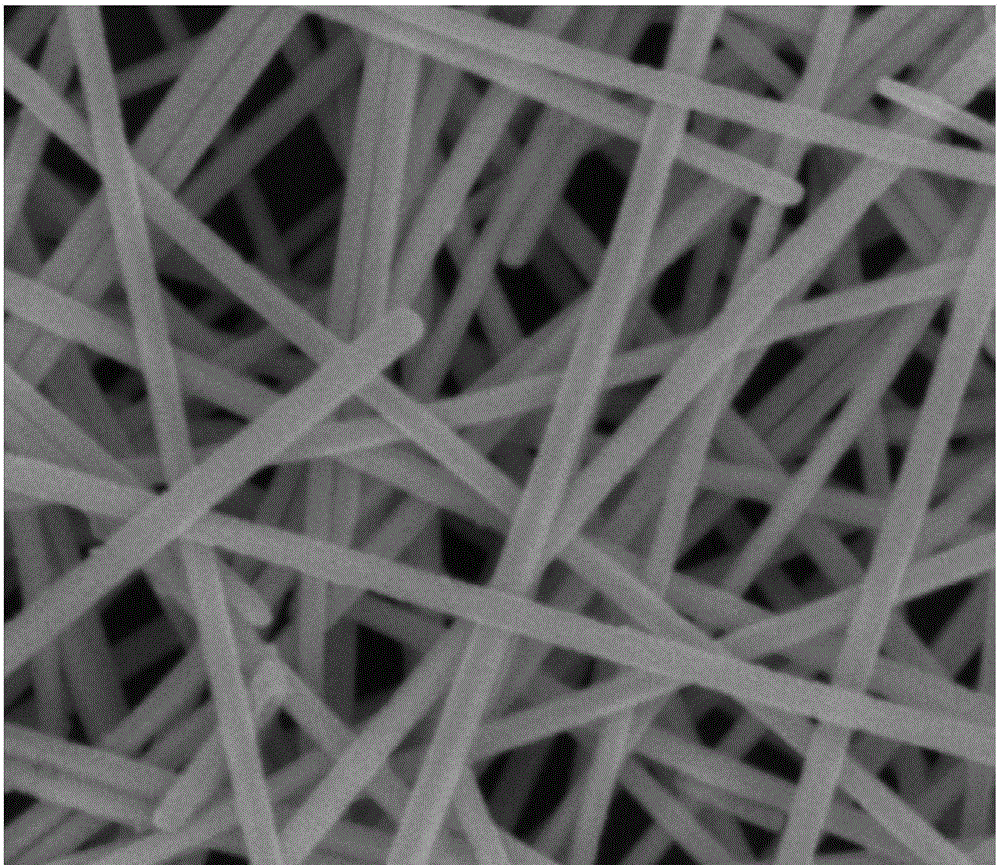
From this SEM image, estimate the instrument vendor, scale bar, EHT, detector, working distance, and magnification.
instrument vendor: FEI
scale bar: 400 nm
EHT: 5 kV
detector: TLD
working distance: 4.8 mm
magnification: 100 K X